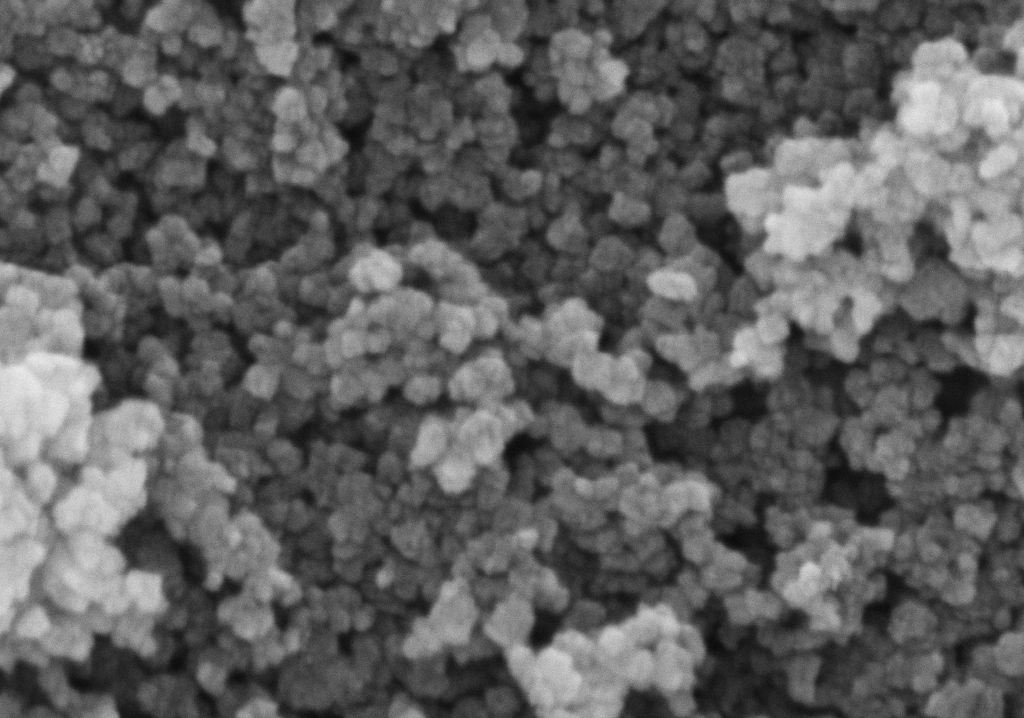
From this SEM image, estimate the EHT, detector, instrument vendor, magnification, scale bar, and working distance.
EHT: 5 kV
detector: InLens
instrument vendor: Zeiss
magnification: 200 K X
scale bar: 100 nm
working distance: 3.9 mm